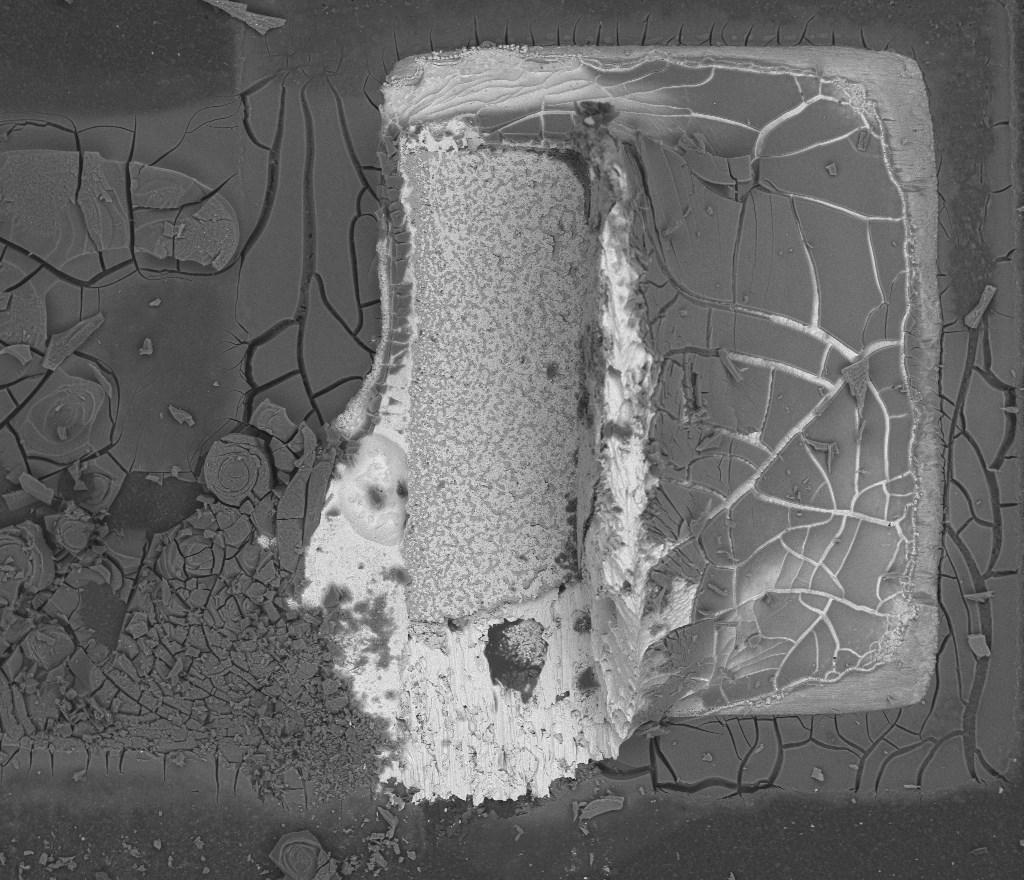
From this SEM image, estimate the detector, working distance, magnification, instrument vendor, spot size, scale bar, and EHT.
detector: BSED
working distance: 11.9 mm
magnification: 0.234 K X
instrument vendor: FEI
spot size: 5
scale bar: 400000 nm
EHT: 20 kV